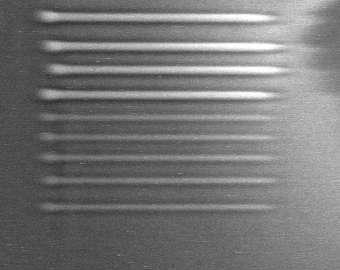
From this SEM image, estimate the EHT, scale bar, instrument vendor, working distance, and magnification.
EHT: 20 kV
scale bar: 25000 nm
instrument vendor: Hitachi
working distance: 16 mm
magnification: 1.2 K X